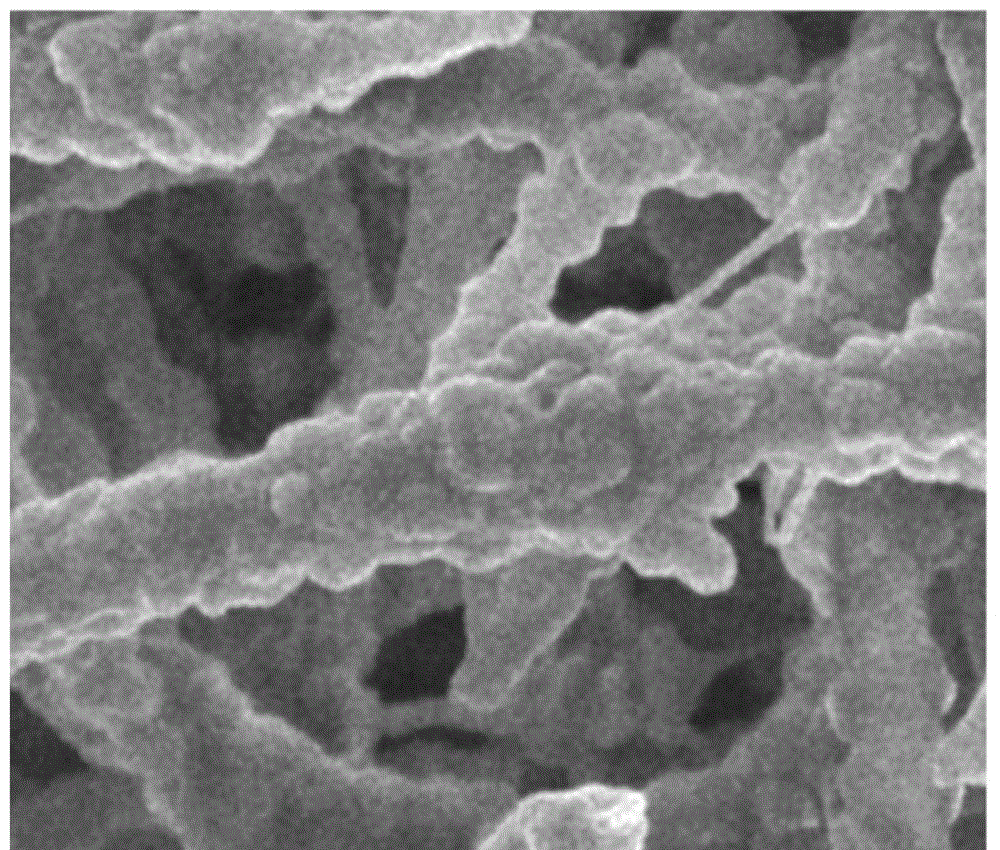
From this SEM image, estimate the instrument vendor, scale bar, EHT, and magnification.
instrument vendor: JEOL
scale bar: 1000 nm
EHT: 5 kV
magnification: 10 K X